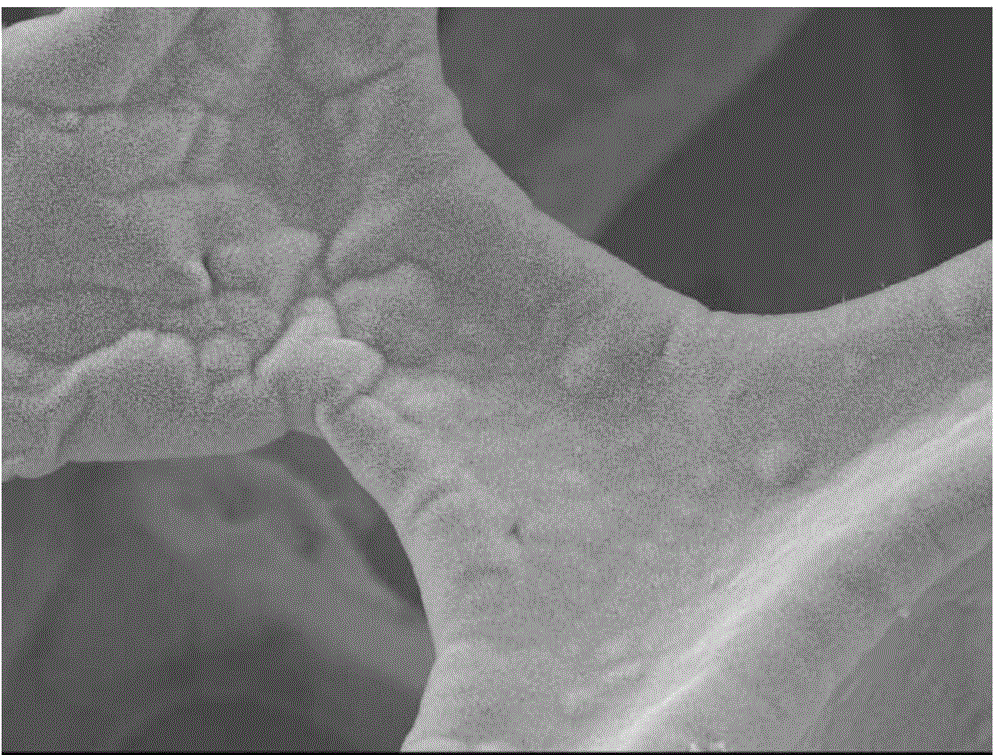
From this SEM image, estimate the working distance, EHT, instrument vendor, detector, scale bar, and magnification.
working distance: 10.3 mm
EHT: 15 kV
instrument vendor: JEOL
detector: SEI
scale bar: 10000 nm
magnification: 0.5 K X